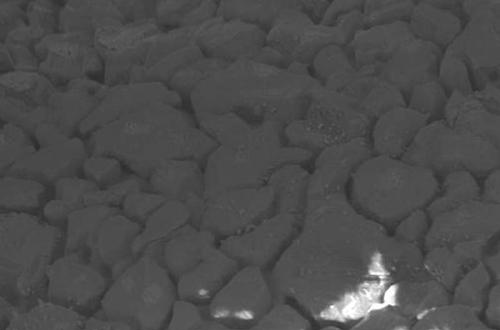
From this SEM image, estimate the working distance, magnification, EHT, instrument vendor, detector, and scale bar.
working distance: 9.3 mm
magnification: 1 K X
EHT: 20 kV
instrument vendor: Zeiss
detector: HDBSD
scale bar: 10000 nm